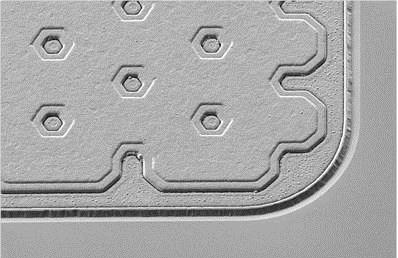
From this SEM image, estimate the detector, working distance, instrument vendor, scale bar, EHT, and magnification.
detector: SE2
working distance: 4.9 mm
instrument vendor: Zeiss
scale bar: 10000 nm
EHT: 3 kV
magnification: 1.64 K X